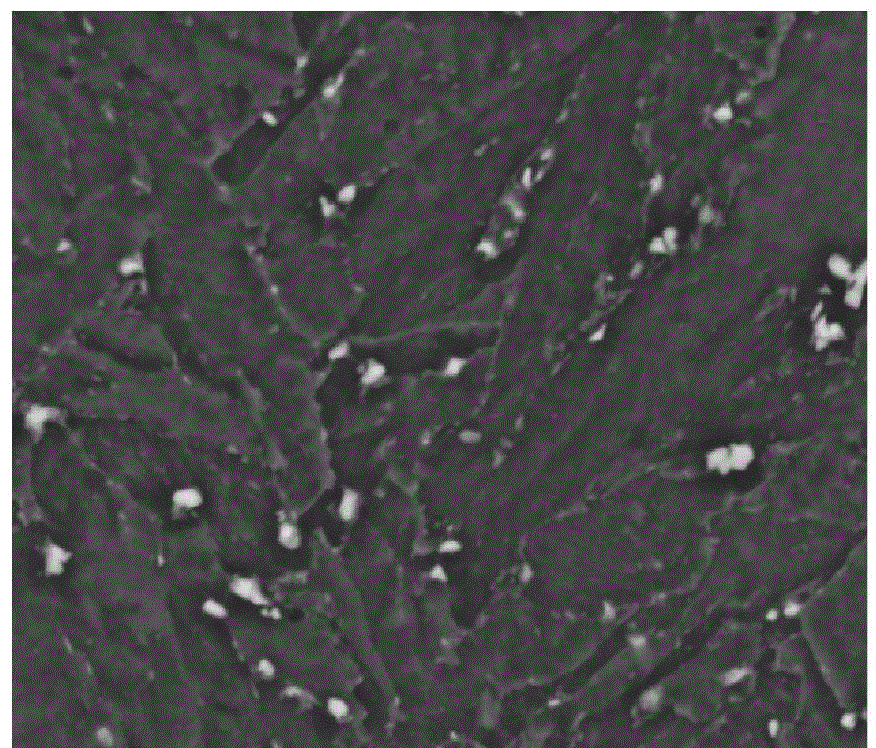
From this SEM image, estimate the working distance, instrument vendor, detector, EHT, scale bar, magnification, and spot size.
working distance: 8 mm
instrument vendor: FEI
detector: SSD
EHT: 20 kV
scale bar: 10000 nm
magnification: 5 K X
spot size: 4.5